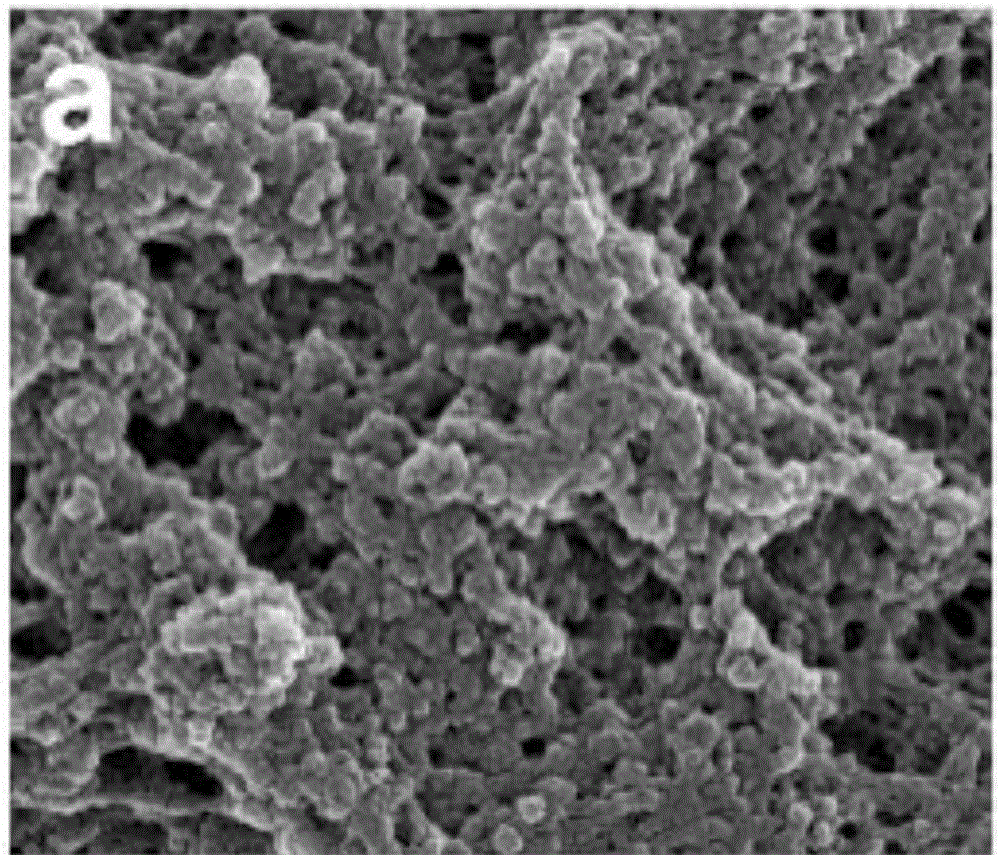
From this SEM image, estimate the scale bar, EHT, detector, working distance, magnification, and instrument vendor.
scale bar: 500 nm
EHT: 10 kV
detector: TLD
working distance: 5.2 mm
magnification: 100 K X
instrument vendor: FEI